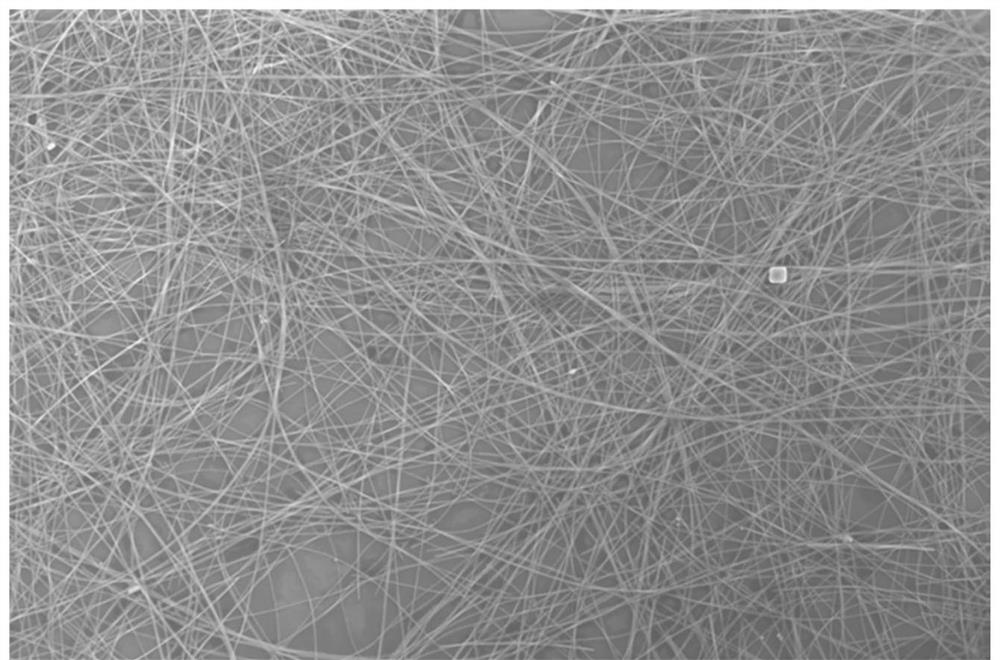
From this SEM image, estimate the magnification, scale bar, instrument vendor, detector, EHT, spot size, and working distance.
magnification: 8 K X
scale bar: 20000 nm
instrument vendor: FEI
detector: TLD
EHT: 10 kV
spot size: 5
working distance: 4.3 mm